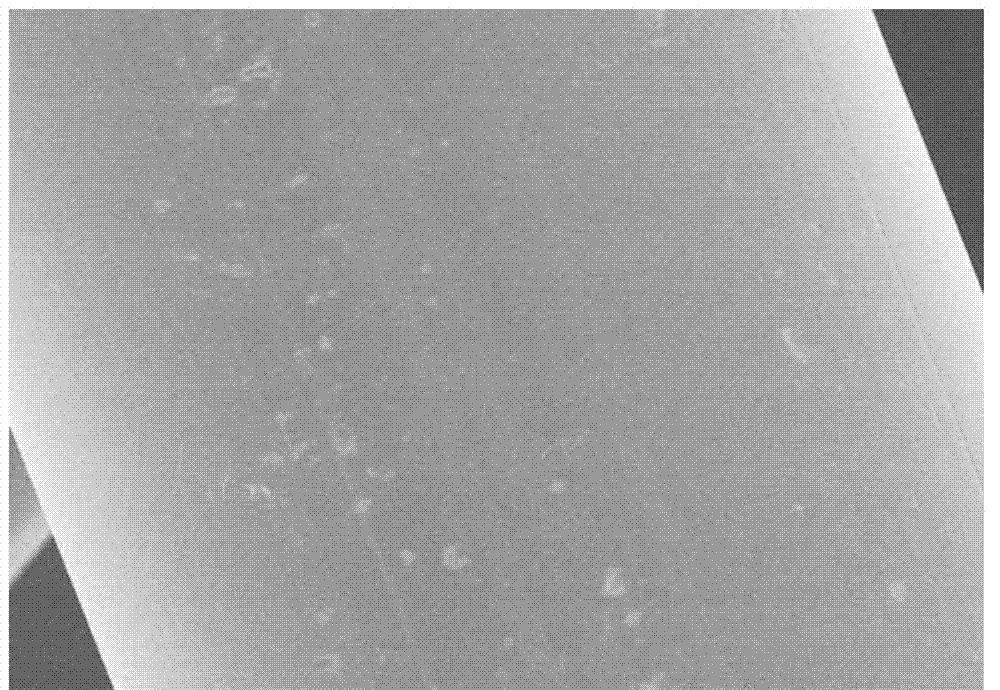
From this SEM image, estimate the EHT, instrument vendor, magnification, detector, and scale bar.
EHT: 5 kV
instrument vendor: Hitachi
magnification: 3.5 K X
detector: SE(M)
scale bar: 10000 nm